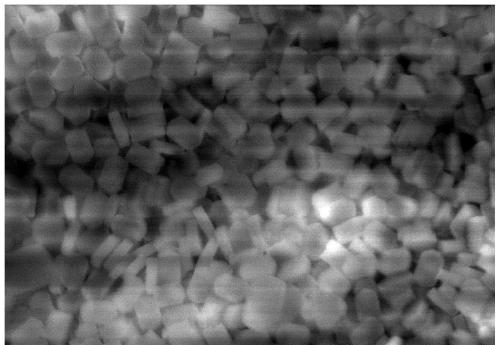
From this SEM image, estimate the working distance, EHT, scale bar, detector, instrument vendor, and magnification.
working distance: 7.1 mm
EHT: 15 kV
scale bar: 5000 nm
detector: SE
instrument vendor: Hitachi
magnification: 10 K X